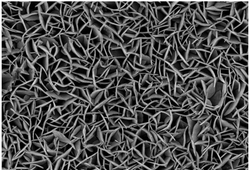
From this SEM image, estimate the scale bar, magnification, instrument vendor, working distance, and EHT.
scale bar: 2000 nm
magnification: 20 K X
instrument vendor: Hitachi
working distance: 2.2 mm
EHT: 3 kV